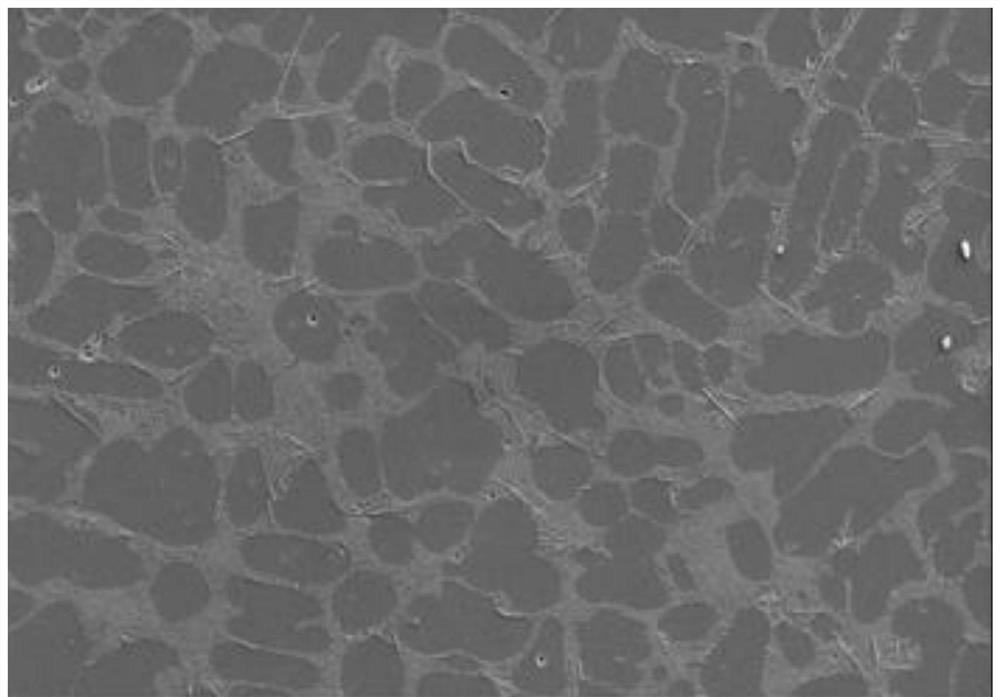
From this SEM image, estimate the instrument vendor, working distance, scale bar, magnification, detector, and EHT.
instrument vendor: Zeiss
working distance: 10.5 mm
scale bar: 40000 nm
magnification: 0.2 K X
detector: SE2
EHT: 20 kV